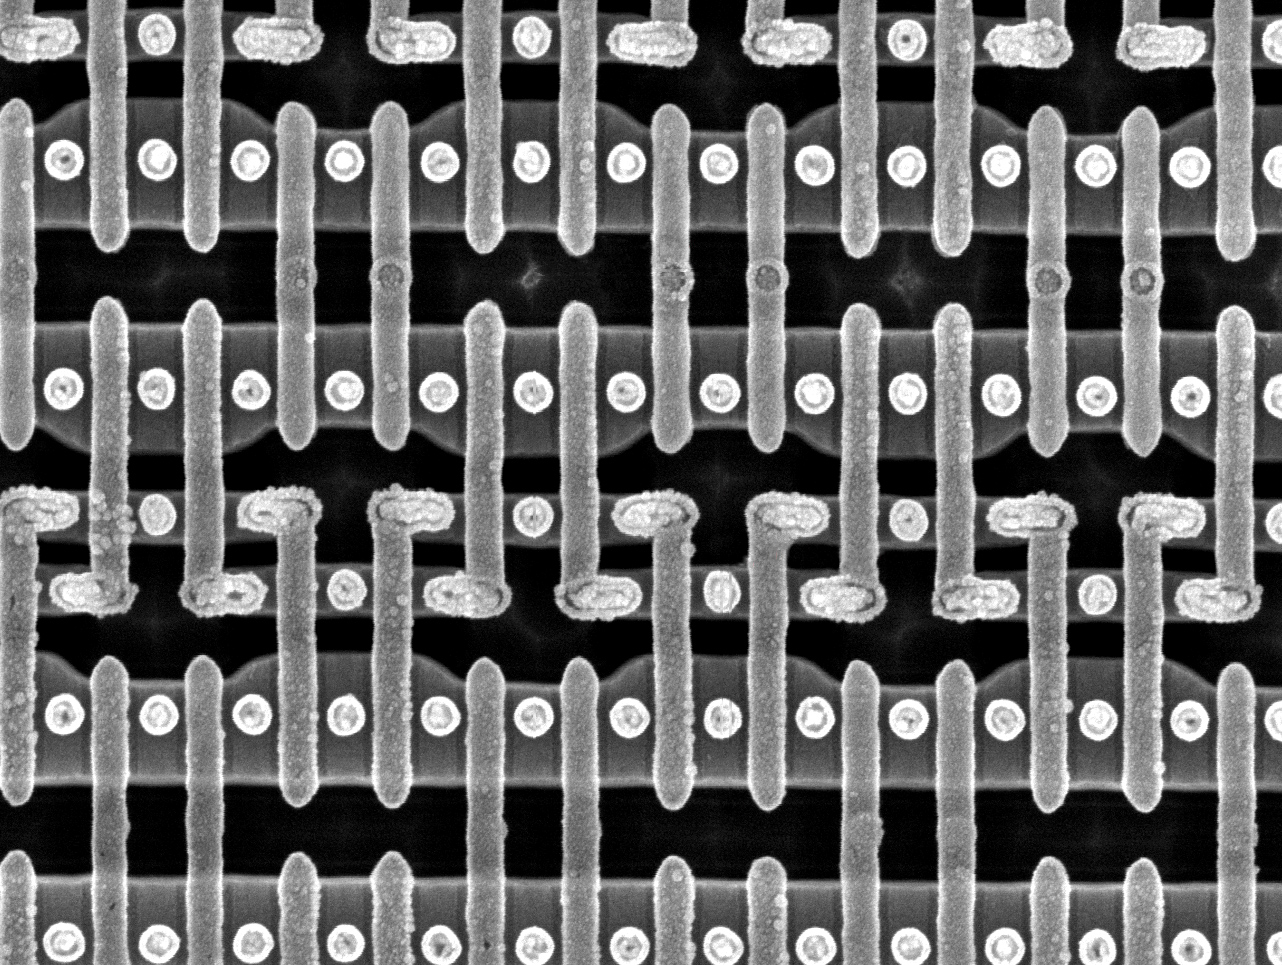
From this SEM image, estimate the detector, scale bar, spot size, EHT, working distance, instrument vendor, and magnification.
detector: TLD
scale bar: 500 nm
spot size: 3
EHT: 10 kV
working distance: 5.3 mm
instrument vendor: FEI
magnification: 50 K X